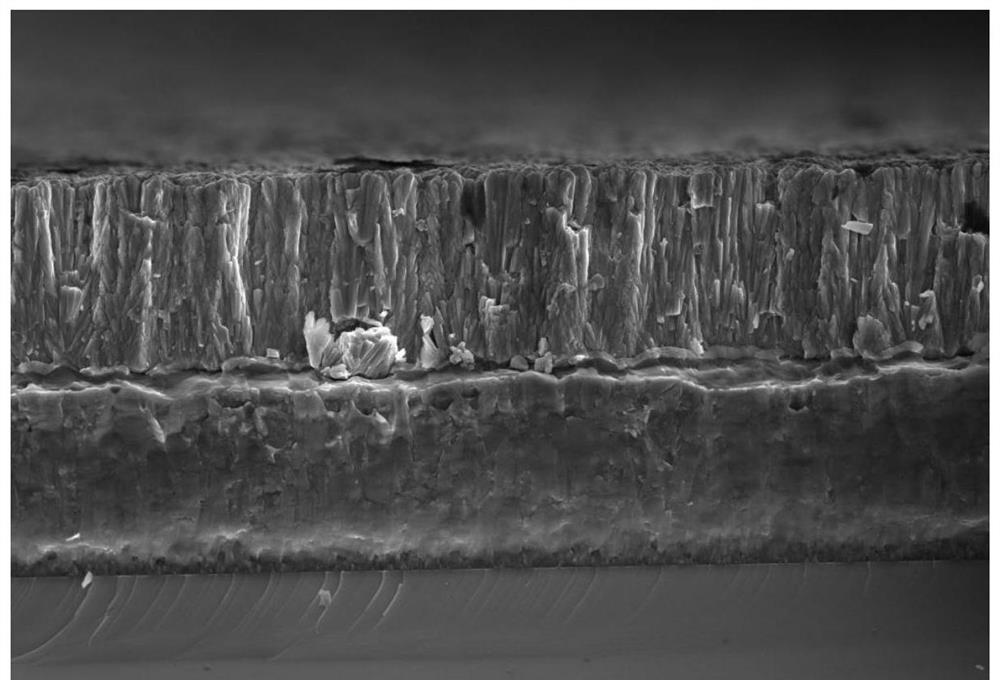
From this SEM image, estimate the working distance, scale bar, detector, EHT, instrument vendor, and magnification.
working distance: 3.4 mm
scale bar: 4000 nm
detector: TLD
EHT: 5 kV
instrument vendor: FEI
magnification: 20 K X